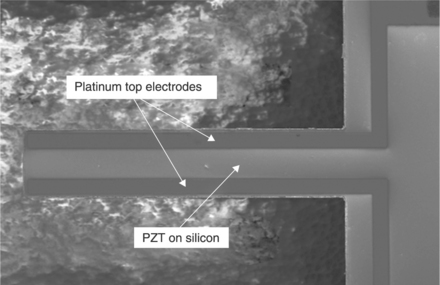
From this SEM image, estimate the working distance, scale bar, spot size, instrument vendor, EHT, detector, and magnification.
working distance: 9.9 mm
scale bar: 100000 nm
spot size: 3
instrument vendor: FEI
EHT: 5 kV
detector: SE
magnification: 0.258 K X